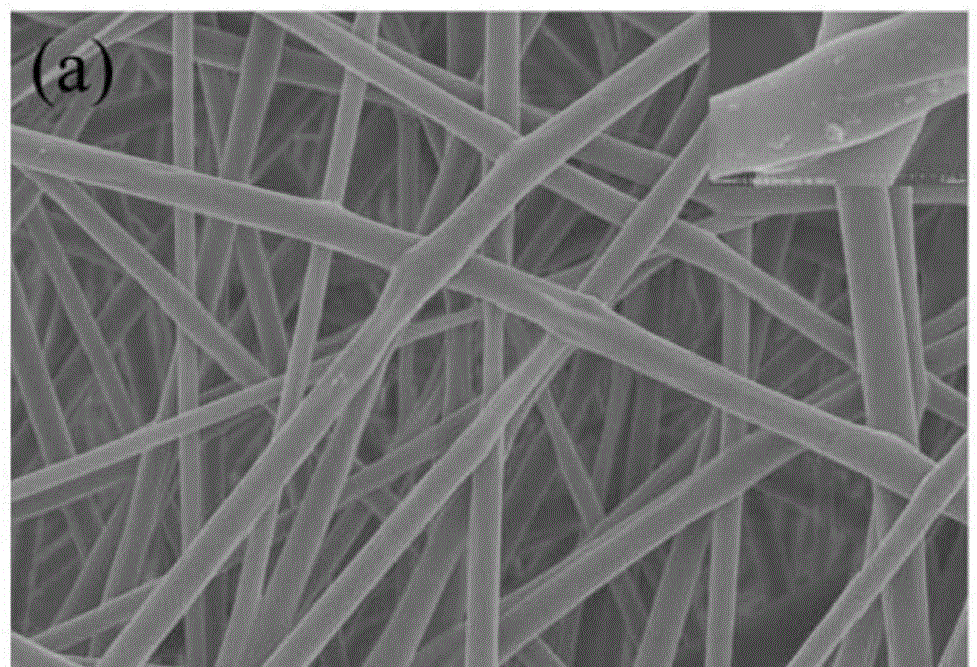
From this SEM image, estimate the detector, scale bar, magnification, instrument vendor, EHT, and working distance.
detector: SE(U)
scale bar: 10000 nm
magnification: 5 K X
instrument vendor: Hitachi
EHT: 5 kV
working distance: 8.2 mm